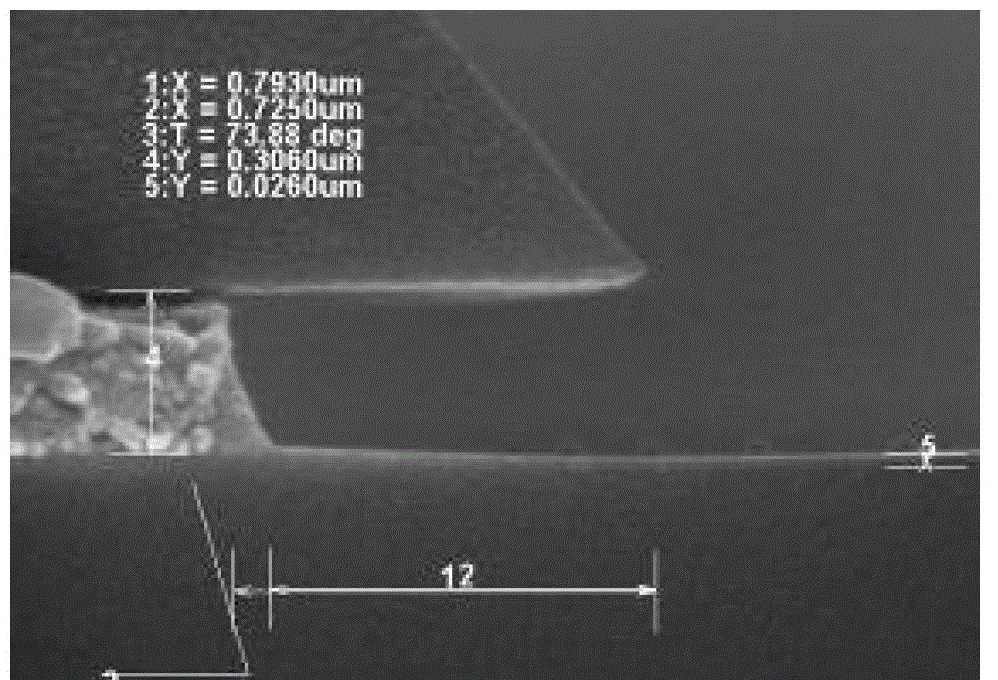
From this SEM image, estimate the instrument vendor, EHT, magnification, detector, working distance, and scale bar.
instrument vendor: Hitachi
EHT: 15 kV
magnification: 70 K X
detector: SE(U)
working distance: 11.7 mm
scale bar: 500 nm